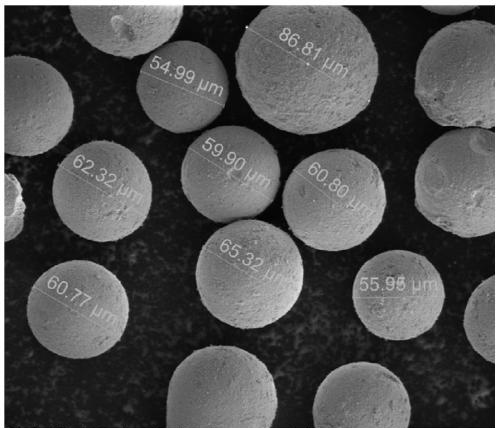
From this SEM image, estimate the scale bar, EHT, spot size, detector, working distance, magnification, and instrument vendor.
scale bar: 50000 nm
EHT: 3 kV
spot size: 3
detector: ETD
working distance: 4.6 mm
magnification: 1 K X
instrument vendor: FEI